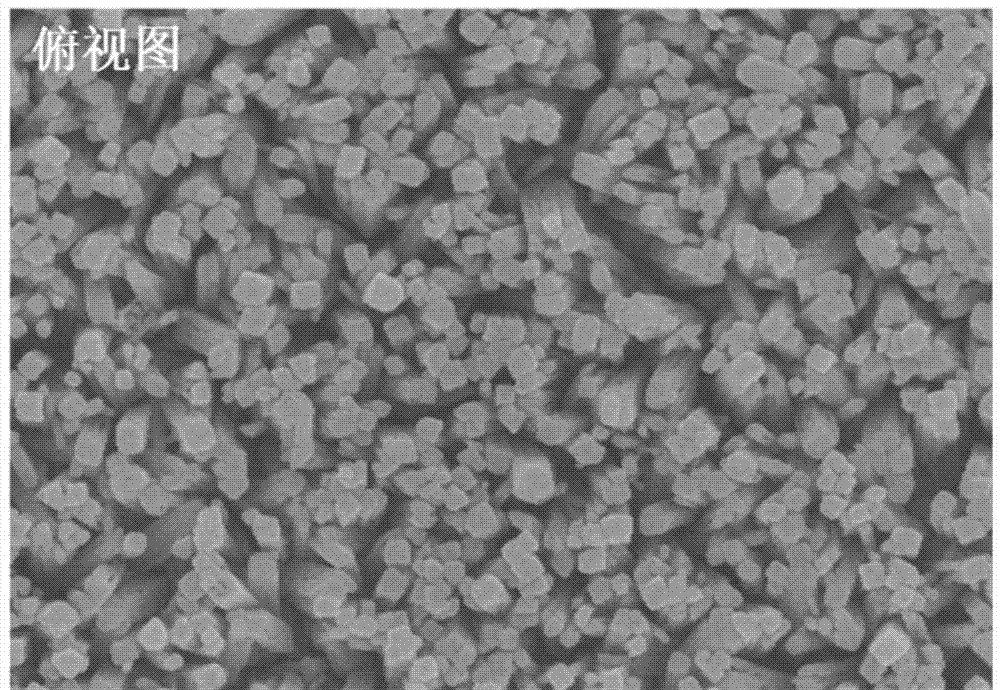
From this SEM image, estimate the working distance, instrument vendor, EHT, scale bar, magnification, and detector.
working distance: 8.4 mm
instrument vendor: Hitachi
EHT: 5 kV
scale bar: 1000 nm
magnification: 50 K X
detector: SE(UL)